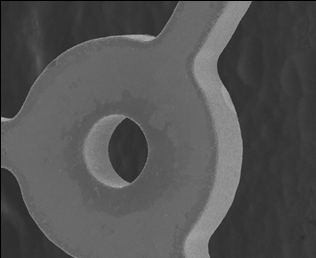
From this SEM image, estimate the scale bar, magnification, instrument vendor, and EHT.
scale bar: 100000 nm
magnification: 0.15 K X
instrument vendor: JEOL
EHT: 20 kV